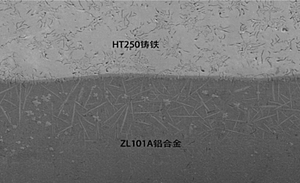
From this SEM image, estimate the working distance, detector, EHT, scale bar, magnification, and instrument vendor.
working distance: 15.4 mm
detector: SE(M)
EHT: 15 kV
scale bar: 200000 nm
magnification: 0.2 K X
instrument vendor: Hitachi